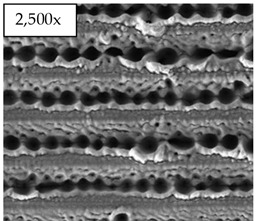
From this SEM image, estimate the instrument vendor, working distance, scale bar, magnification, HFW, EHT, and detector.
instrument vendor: FEI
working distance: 7 mm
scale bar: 40000 nm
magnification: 2.5 K X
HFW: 119 µm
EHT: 30 kV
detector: ETD